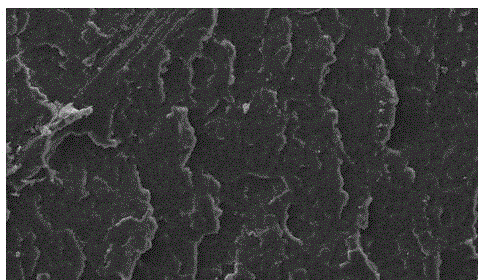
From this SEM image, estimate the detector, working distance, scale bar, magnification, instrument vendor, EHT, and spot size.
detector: SE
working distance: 2.8 mm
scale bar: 100000 nm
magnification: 0.2 K X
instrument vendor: FEI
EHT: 20 kV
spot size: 4